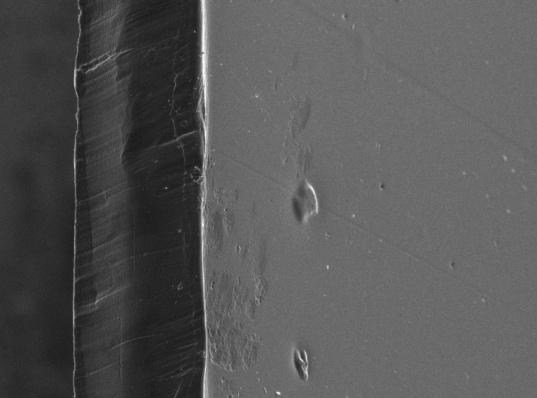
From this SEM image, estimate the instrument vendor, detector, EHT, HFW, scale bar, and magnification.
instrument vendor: Tescan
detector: SE Detector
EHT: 5 kV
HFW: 271.46 µm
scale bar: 100000 nm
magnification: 1 K X